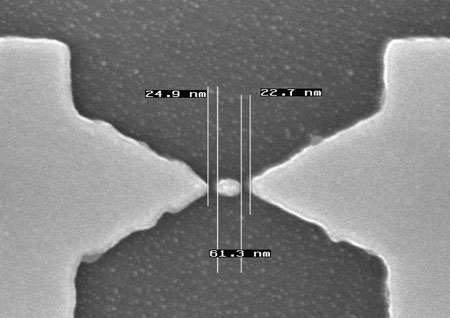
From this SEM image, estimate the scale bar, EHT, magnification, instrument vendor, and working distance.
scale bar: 200 nm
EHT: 10 kV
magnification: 100 K X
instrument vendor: other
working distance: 4 mm